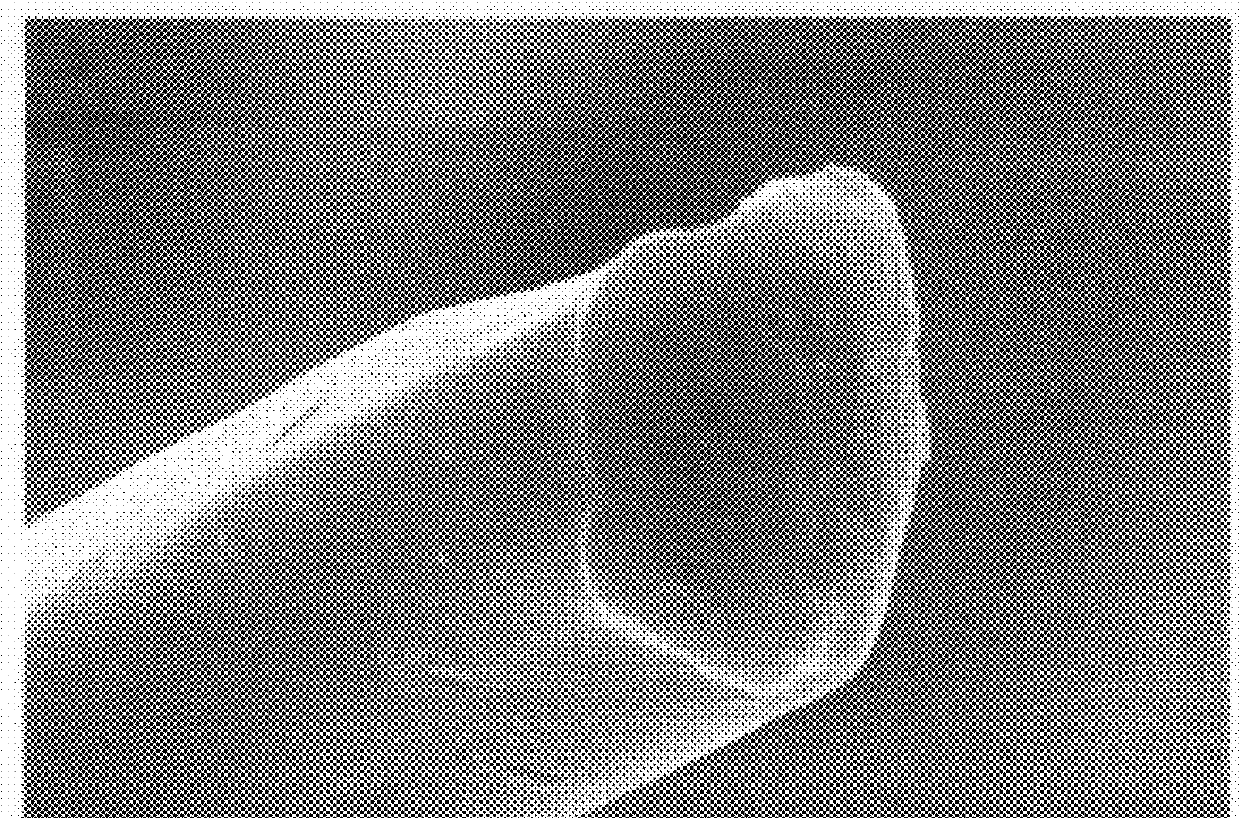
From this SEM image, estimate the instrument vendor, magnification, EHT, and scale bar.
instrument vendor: JEOL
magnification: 3 K X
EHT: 20 kV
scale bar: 5000 nm